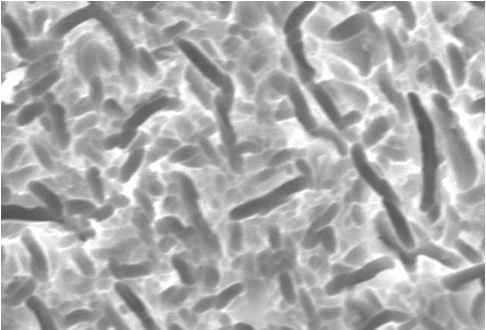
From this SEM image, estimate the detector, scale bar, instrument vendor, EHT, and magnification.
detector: SEI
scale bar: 5000 nm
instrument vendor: JEOL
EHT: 20 kV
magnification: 3 K X